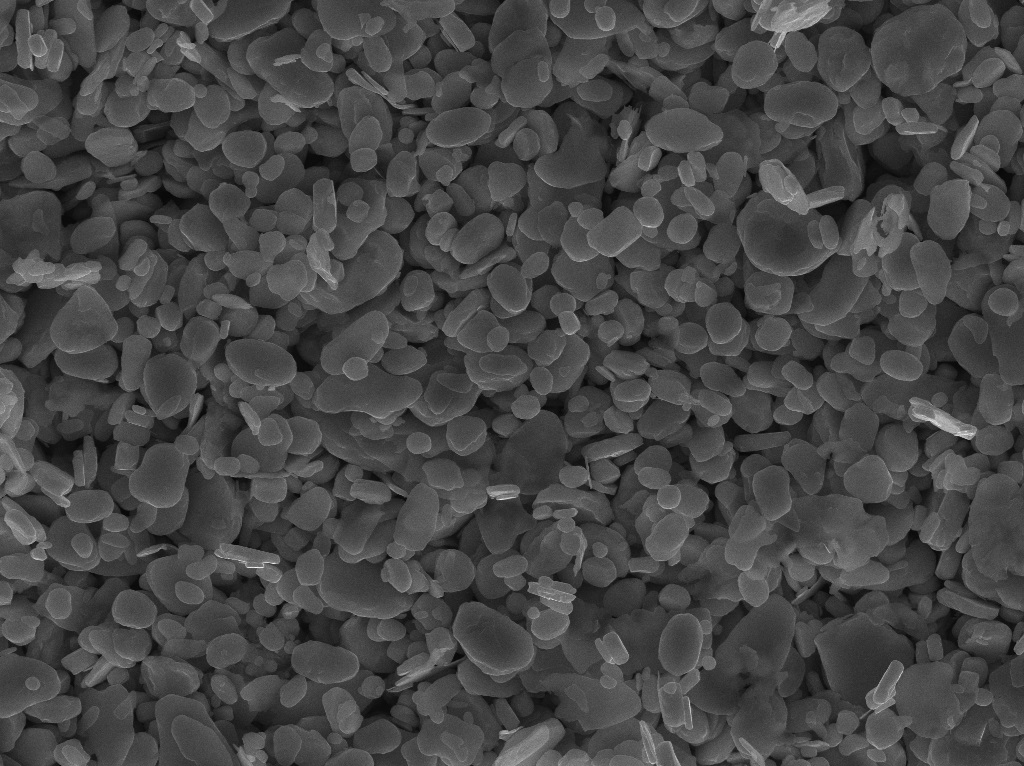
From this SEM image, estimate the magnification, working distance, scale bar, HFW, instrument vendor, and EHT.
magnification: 5 K X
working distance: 10.13 mm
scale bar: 10000 nm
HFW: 55.2 µm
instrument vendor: Tescan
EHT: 30 kV